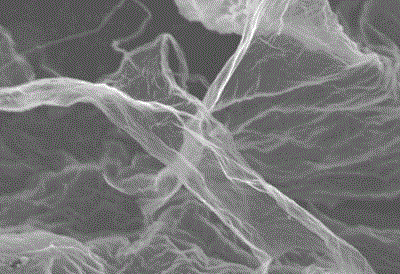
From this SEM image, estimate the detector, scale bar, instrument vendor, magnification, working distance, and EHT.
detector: SEI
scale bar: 1000 nm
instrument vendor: JEOL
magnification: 5 K X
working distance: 8.4 mm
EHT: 8 kV